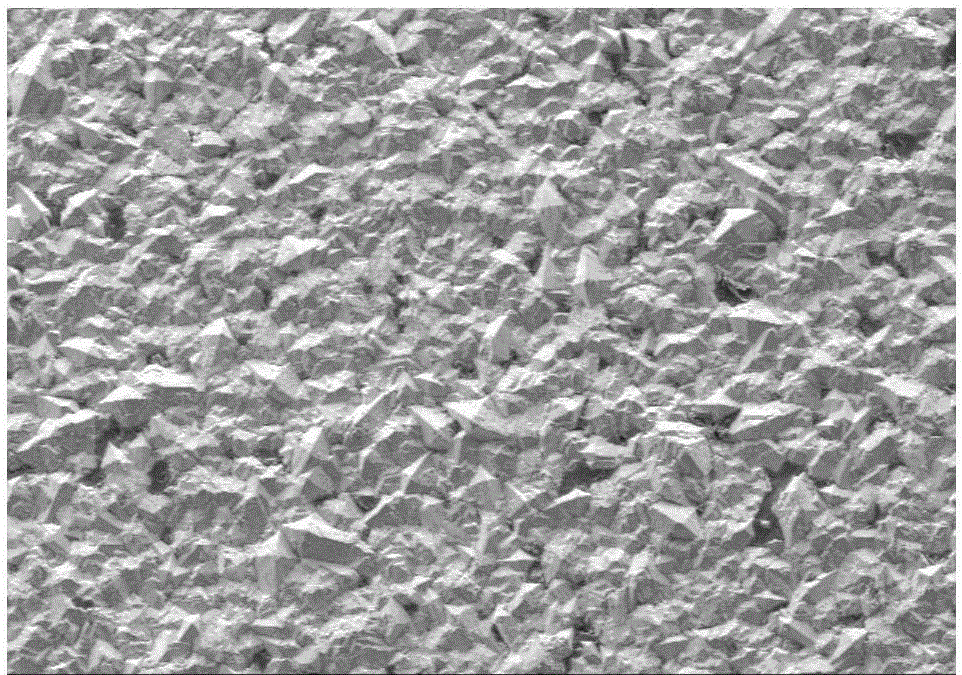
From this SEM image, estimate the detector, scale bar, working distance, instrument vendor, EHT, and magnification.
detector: SE(M)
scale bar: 500000 nm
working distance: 12.8 mm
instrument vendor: Hitachi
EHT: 15 kV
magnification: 0.1 K X